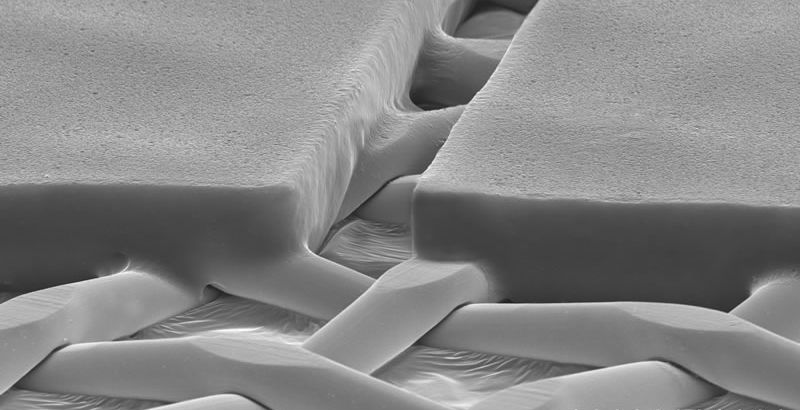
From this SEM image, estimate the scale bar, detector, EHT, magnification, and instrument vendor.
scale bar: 50000 nm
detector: SE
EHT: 30 kV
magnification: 0.65 K X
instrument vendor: Hitachi